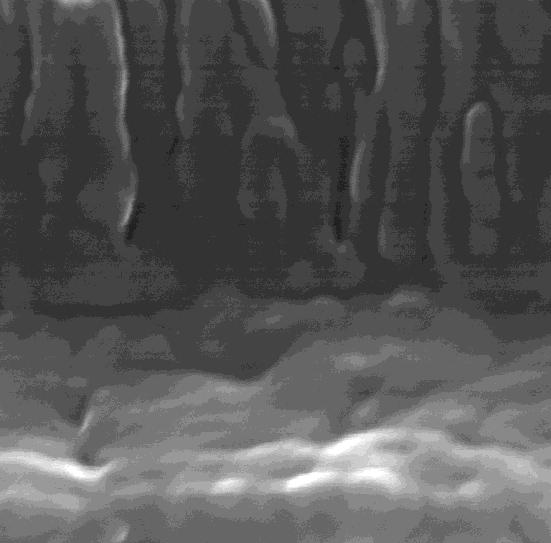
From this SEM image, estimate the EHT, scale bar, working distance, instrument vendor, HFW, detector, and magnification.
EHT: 20 kV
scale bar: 100 nm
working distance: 2.494 mm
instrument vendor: Tescan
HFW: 0.543 µm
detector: InBeam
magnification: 398.99 K X